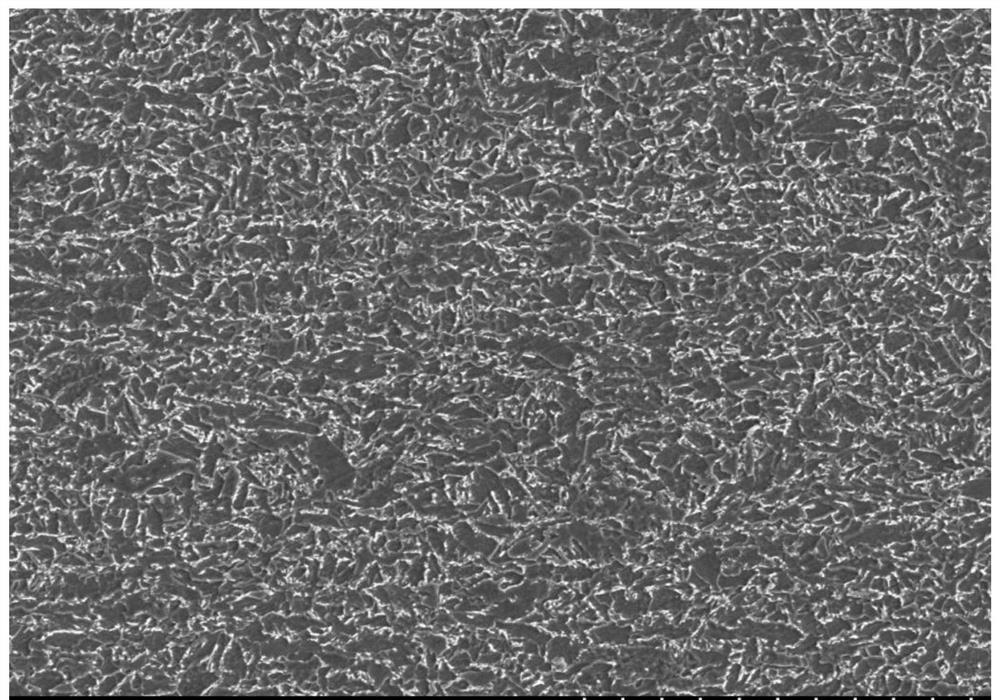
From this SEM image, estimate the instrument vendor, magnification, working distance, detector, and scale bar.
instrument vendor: Hitachi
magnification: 1 K X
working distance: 9.5 mm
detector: SE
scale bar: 50000 nm